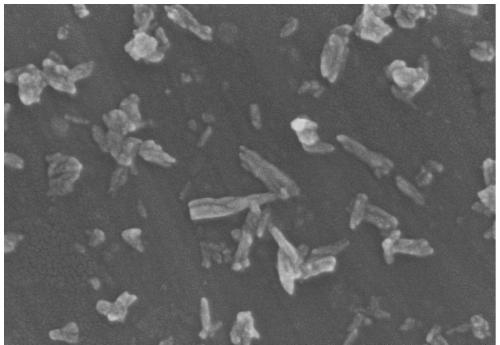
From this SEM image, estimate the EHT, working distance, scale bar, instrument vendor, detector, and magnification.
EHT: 5 kV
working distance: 7.8 mm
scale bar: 500 nm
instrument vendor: Hitachi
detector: SE(M)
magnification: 100 K X